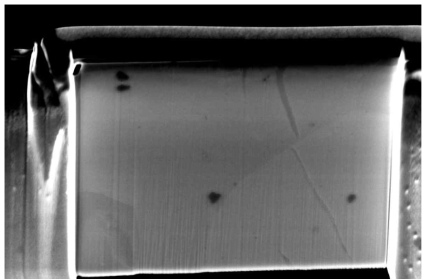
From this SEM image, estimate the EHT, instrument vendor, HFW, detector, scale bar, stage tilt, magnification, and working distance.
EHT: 5 kV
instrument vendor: FEI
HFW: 10.6 µm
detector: TLD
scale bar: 3000 nm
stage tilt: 53°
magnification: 12 K X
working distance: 4 mm